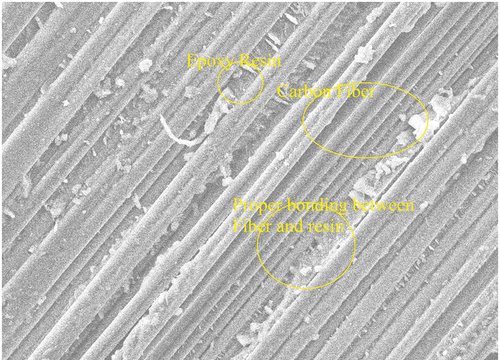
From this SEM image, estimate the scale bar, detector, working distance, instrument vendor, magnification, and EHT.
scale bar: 20000 nm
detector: SED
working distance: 14.5 mm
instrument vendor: JEOL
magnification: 0.55 K X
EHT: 15 kV